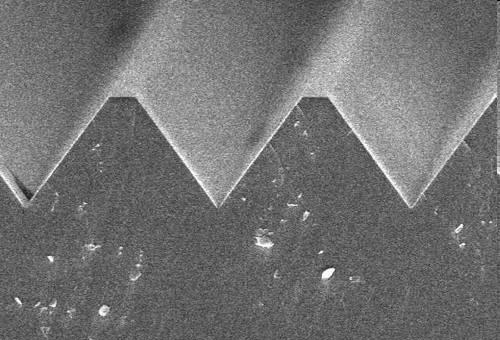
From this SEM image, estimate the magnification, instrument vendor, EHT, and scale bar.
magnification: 1.8 K X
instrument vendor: Hitachi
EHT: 15 kV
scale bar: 16700 nm